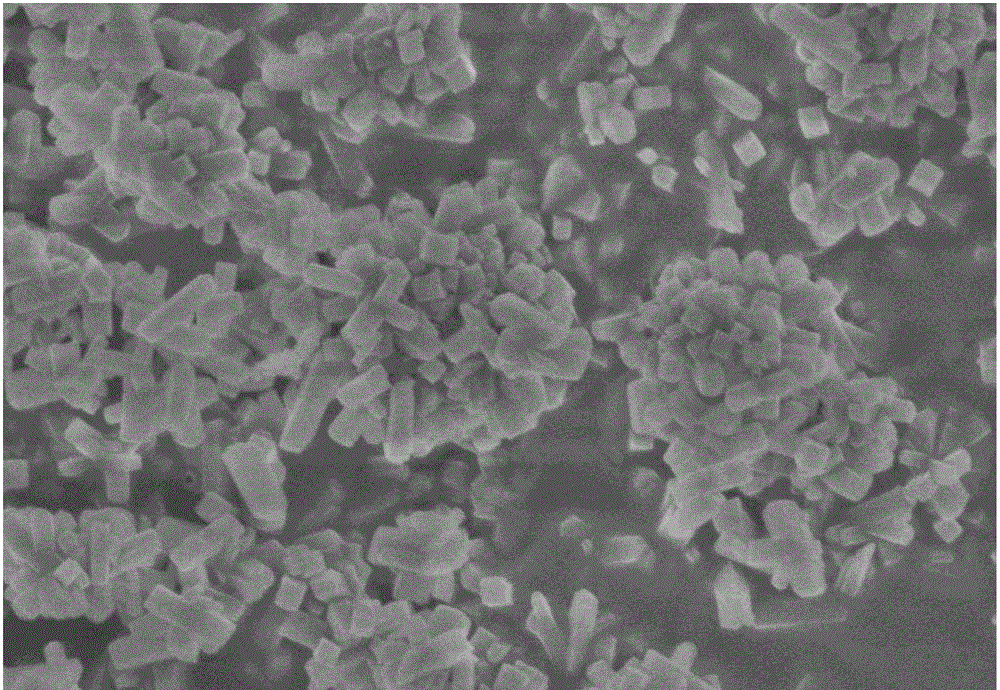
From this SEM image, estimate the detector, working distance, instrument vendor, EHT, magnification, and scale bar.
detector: SE(U)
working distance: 8.2 mm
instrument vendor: Hitachi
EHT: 3 kV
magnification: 30 K X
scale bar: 1000 nm